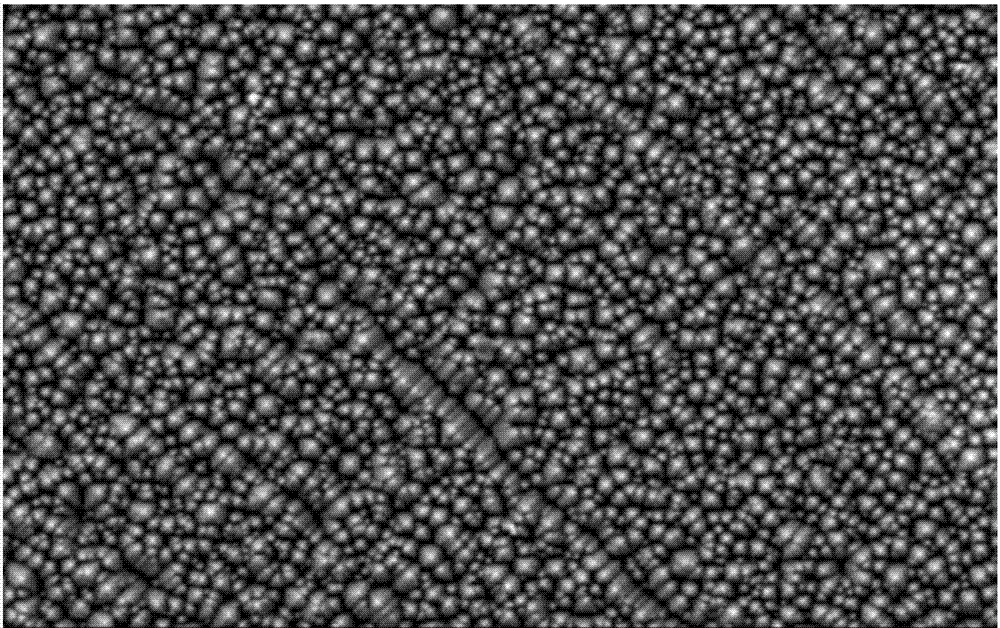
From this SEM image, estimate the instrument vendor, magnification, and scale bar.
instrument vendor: JEOL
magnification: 1.5 K X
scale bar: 10000 nm